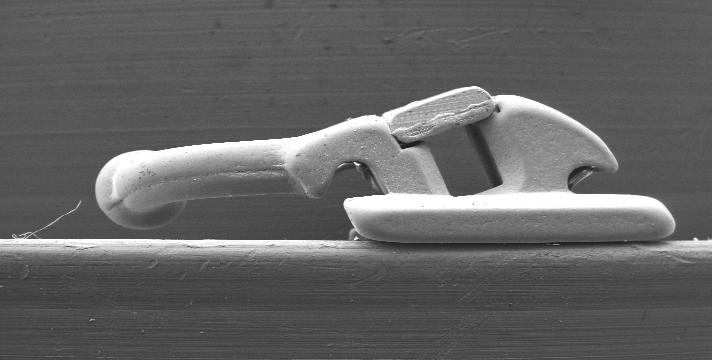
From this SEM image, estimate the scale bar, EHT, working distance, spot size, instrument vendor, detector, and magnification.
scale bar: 2000000 nm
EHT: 20 kV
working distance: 18.9 mm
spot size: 7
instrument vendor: FEI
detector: SE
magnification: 0.035 K X